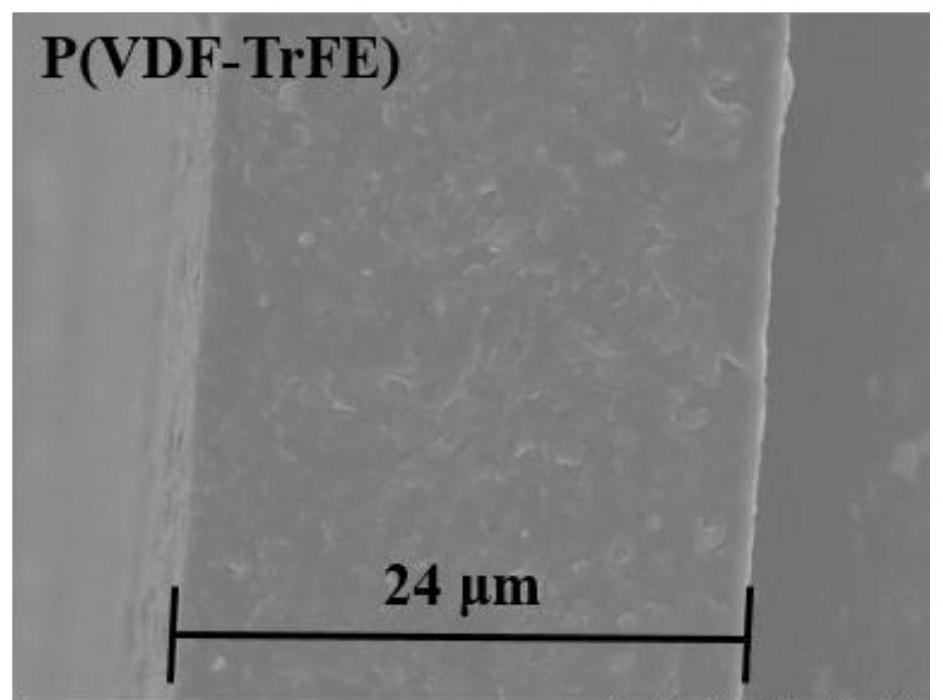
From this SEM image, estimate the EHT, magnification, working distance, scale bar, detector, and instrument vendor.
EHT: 7 kV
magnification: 4 K X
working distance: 8.7 mm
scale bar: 10000 nm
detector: SE(UL)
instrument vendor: Hitachi